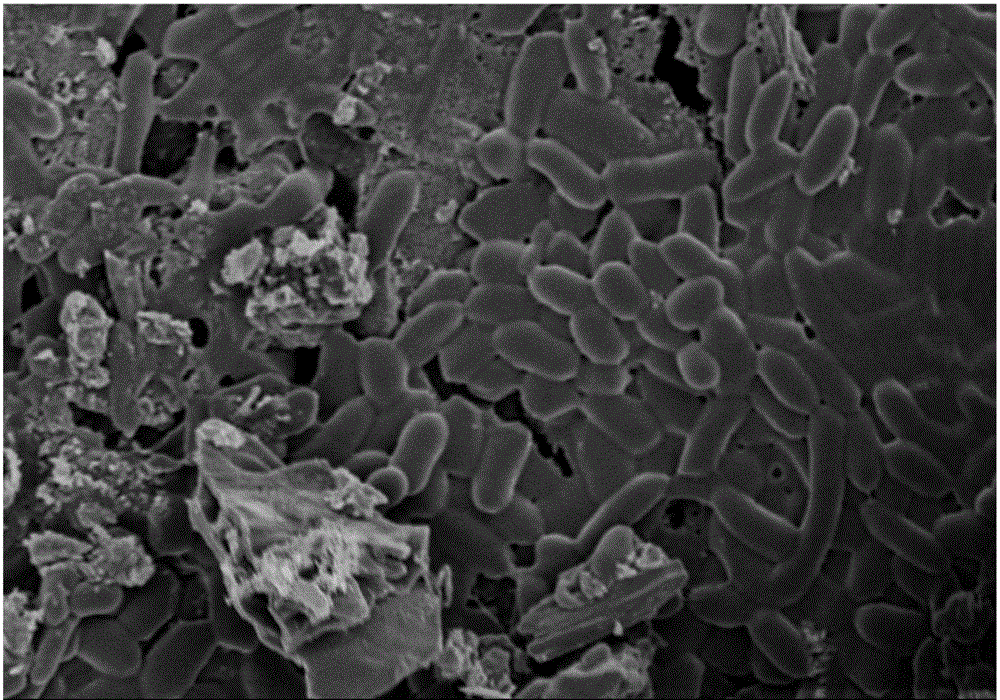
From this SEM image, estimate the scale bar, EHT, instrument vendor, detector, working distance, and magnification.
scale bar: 5000 nm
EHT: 2 kV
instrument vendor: Hitachi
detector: SE(M)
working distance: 6 mm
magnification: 10 K X